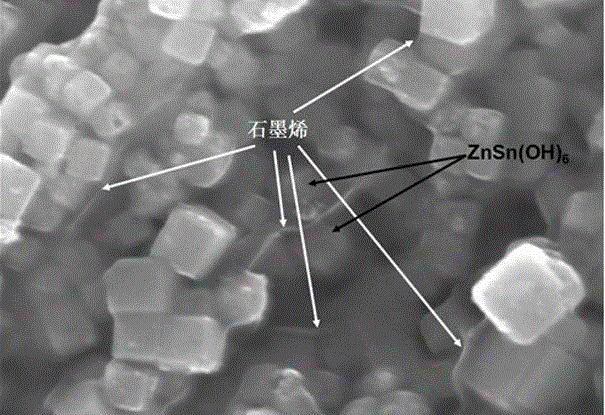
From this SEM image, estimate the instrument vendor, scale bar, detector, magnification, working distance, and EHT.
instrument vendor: Hitachi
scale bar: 500 nm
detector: SE(UL)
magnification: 110 K X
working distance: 8.6 mm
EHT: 10 kV